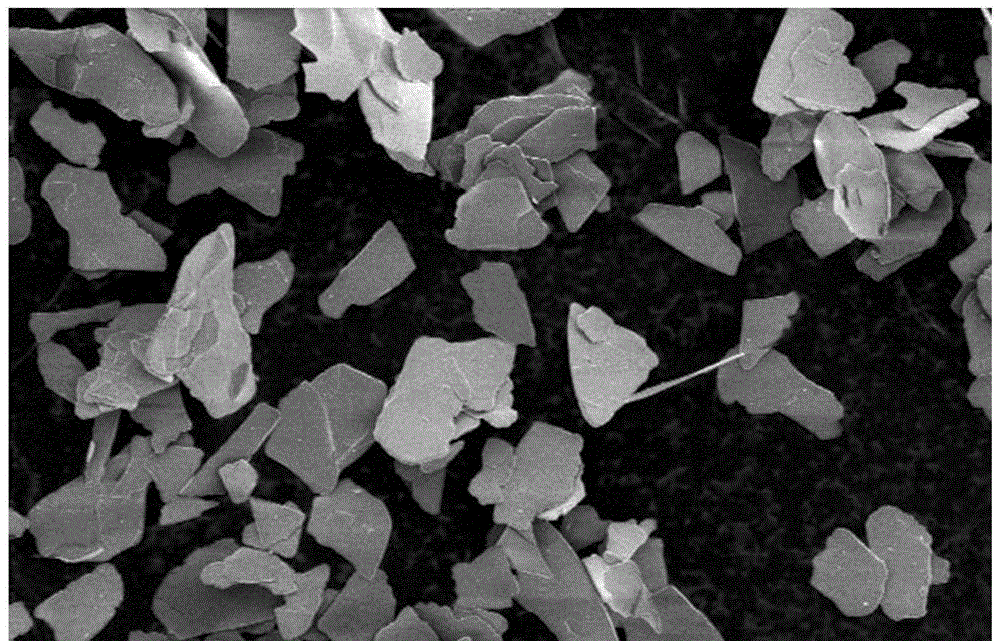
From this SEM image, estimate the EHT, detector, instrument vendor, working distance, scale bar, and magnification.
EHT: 5 kV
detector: SE(M)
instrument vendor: Hitachi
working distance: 5.2 mm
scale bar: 100000 nm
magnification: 0.5 K X